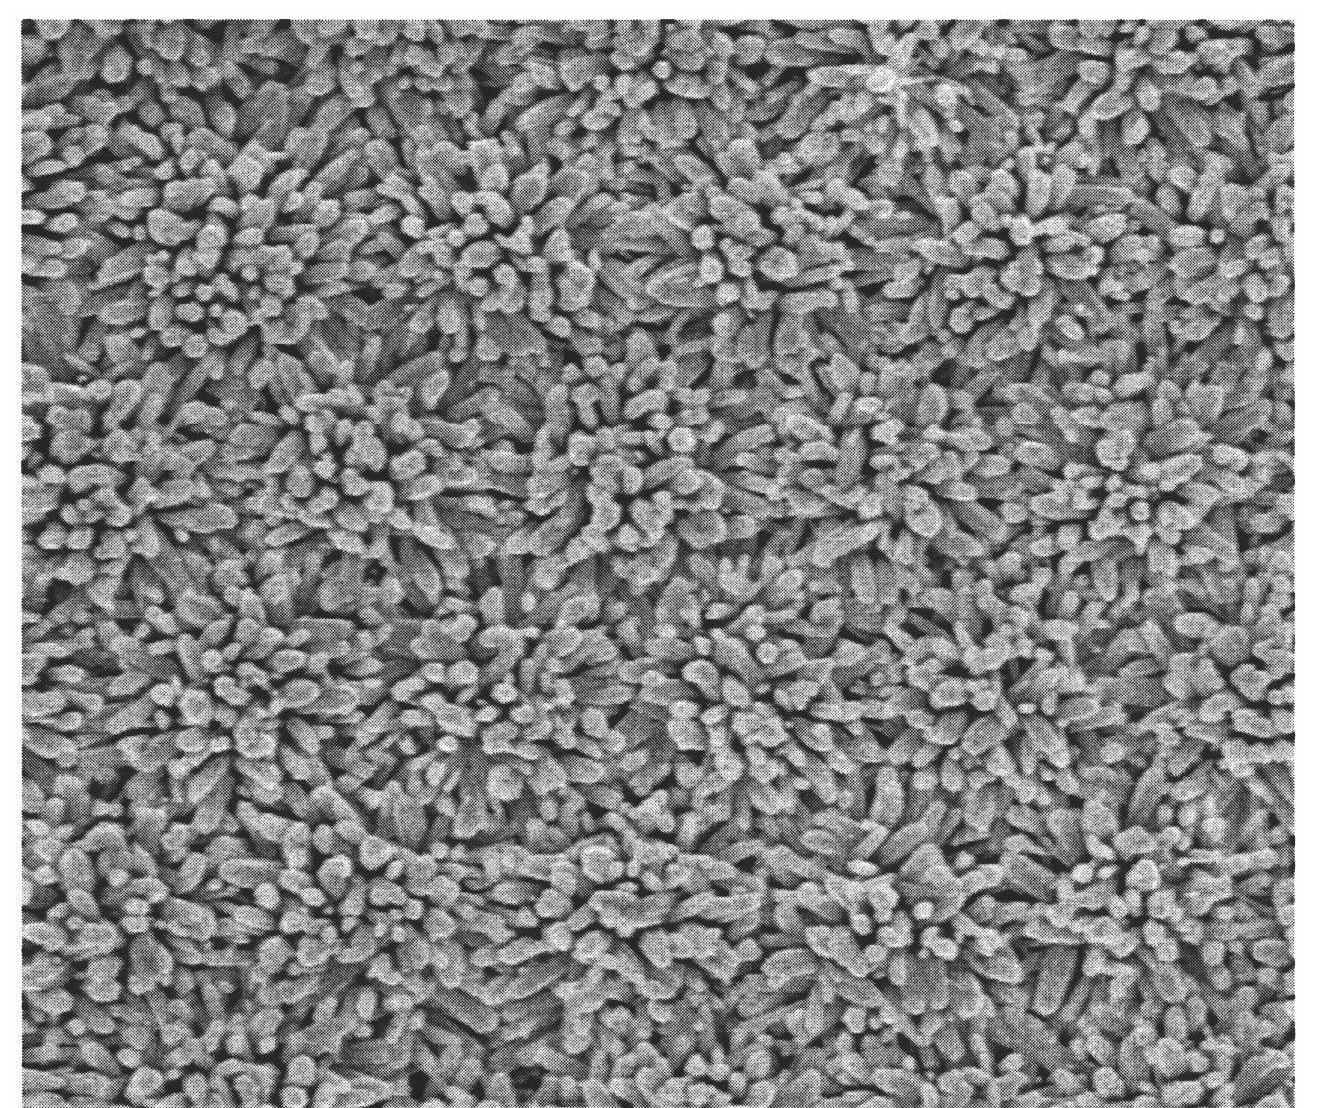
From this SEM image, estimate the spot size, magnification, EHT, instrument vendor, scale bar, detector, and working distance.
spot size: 3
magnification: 10 K X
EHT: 5 kV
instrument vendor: FEI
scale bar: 2000 nm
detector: TLD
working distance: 5.4 mm